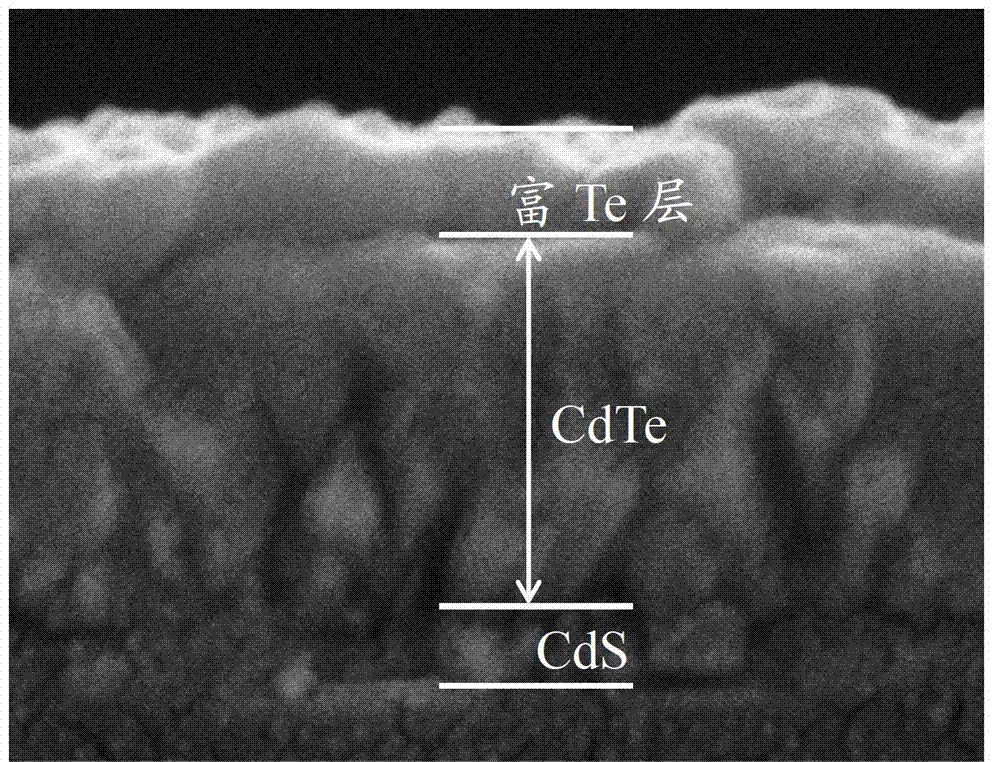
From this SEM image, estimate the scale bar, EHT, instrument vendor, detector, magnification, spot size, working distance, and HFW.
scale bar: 1000 nm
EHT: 10 kV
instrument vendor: FEI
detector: ETD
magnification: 40 K X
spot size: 2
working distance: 4.9 mm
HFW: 3.73 µm